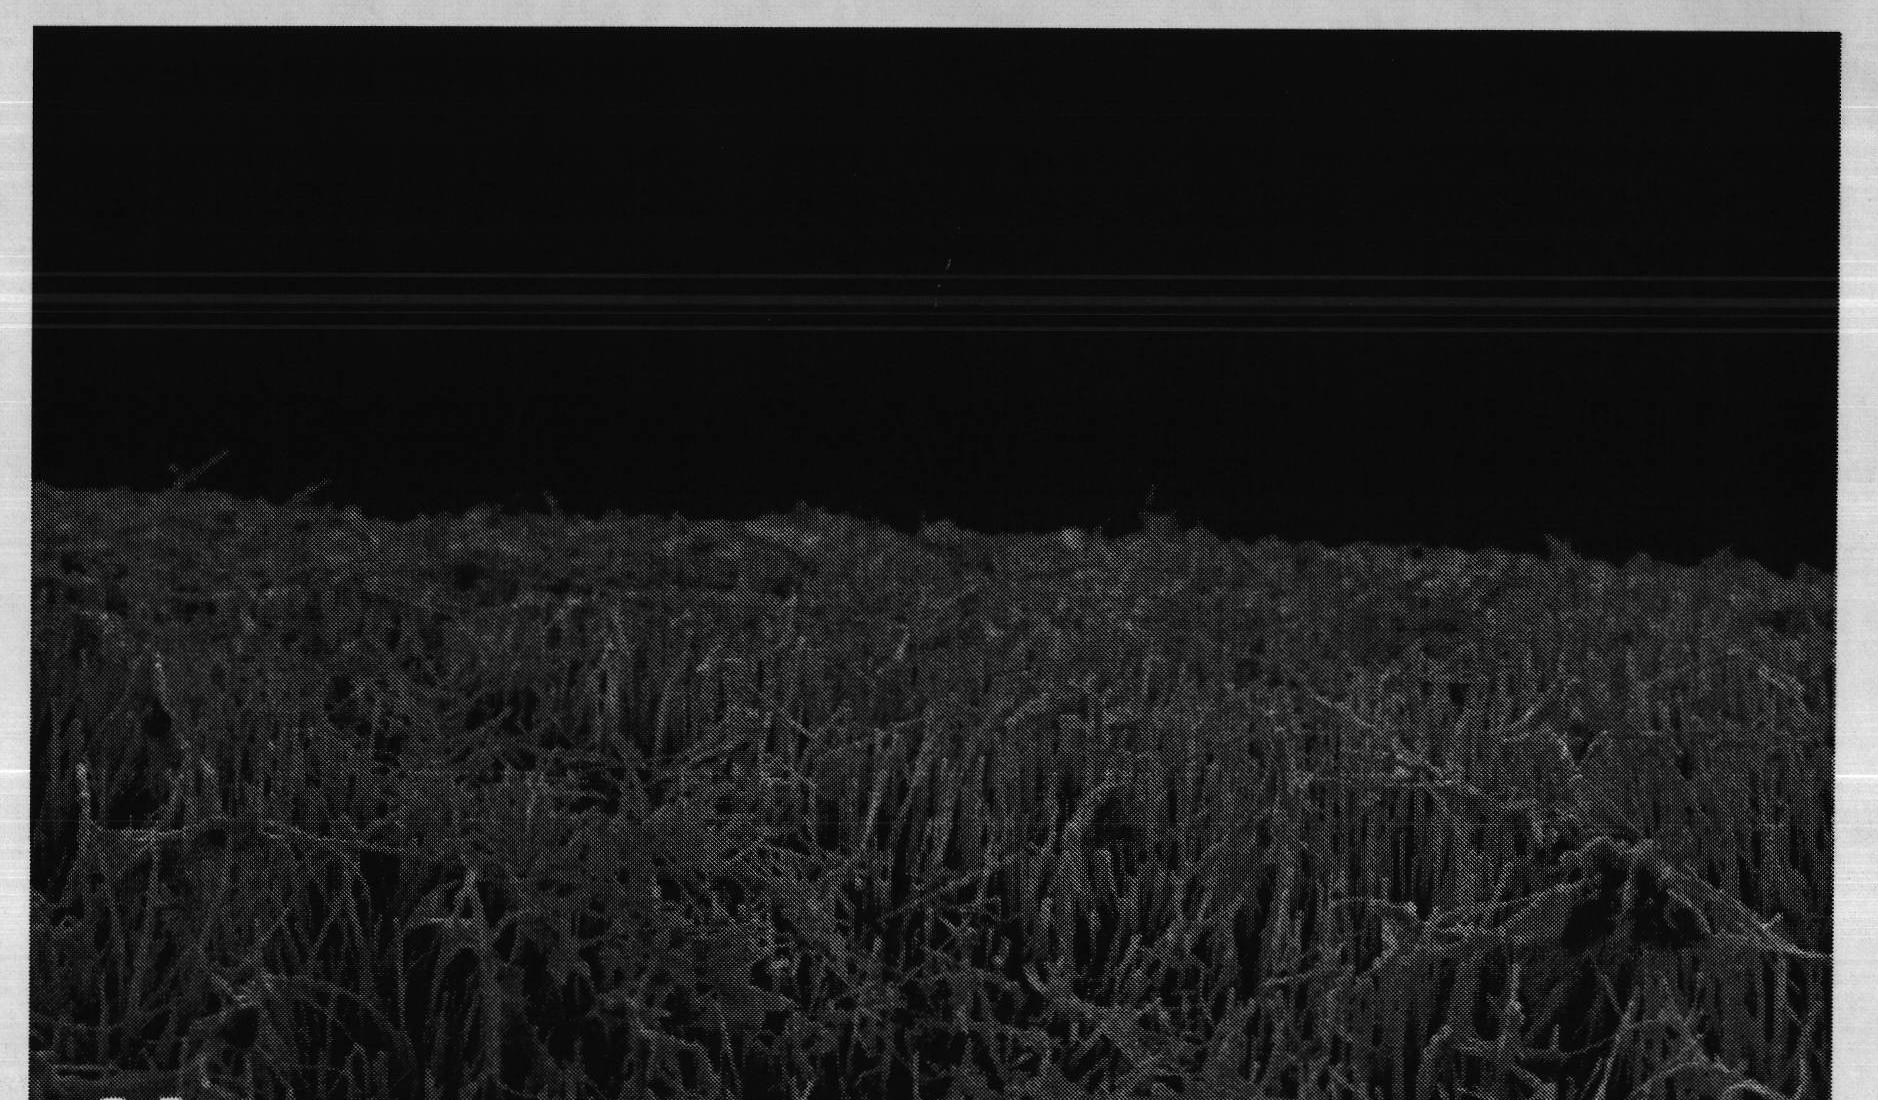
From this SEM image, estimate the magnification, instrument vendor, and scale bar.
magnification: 1 K X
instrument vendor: other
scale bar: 10000 nm